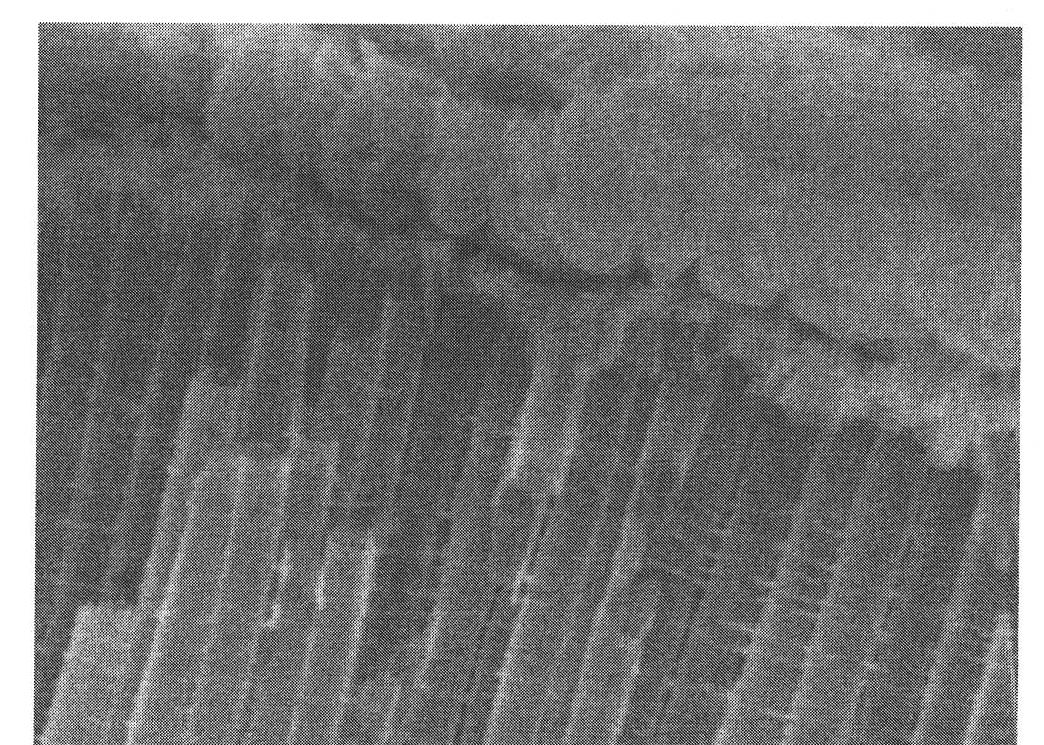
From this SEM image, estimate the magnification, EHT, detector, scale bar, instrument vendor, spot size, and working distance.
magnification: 70.652 K X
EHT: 5 kV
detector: TLD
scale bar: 200 nm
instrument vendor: FEI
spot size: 3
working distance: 4.5 mm